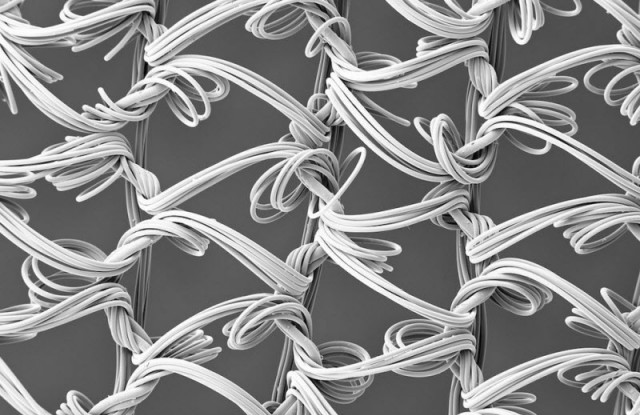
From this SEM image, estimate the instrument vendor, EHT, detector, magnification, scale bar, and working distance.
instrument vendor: Zeiss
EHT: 5 kV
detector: SE2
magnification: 0.05 K X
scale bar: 100000 nm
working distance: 19.4 mm